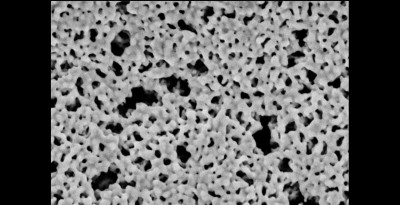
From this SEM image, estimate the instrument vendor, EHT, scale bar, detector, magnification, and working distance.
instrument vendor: Zeiss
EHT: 20 kV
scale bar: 1000 nm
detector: SE1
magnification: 19.76 K X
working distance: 16 mm